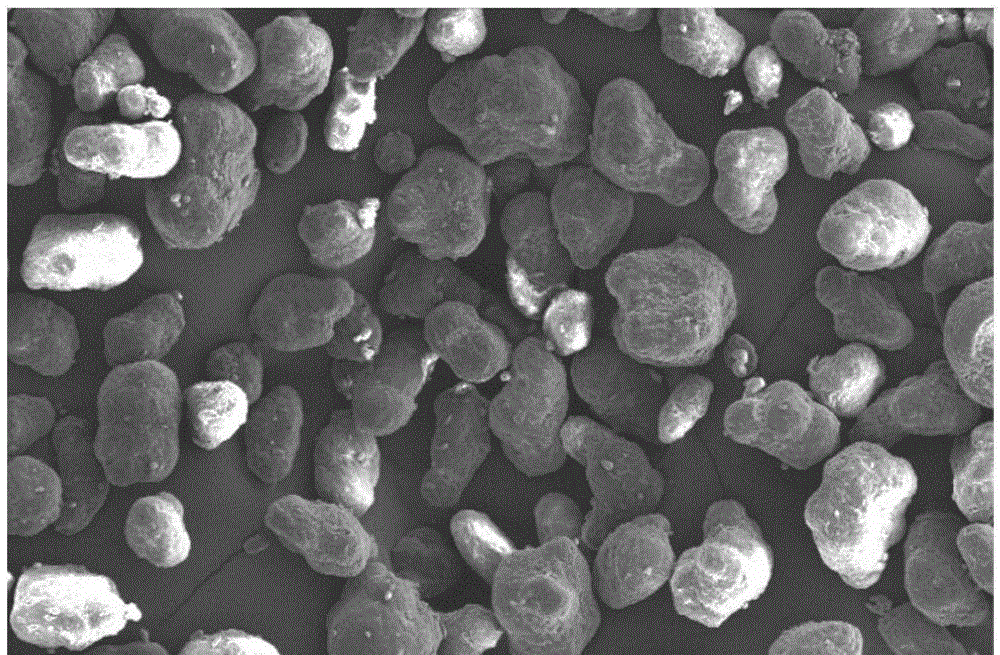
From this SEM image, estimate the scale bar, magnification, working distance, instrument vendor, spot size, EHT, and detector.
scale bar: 100000 nm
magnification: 0.2 K X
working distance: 11.7 mm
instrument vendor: FEI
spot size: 3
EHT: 10 kV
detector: SE